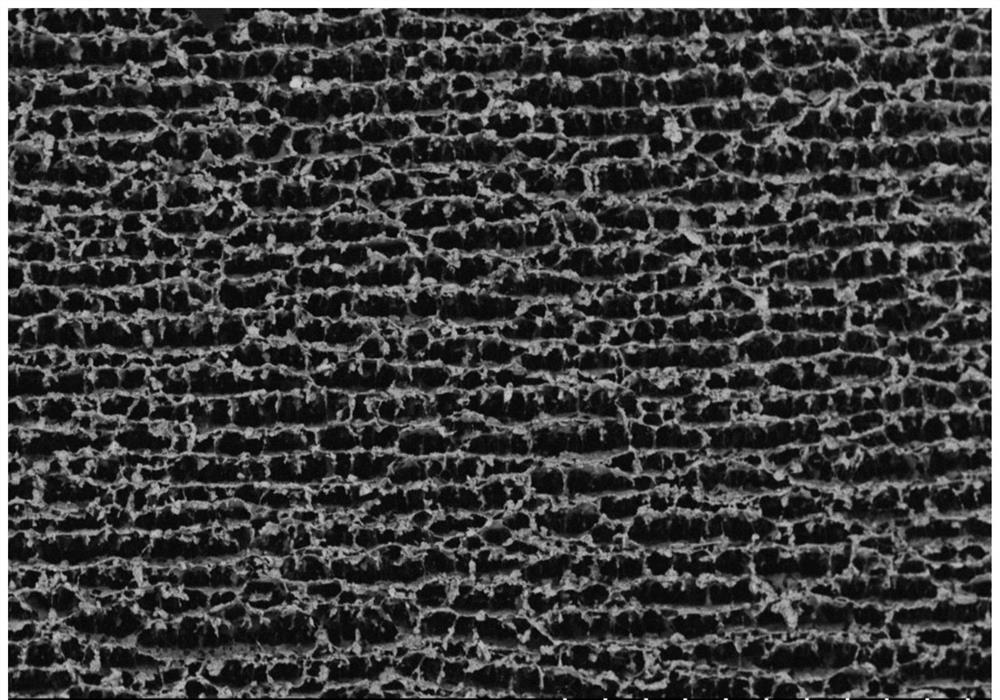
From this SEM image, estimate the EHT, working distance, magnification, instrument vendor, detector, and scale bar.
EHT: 5 kV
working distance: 8.2 mm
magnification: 0.13 K X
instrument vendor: Hitachi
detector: BSE-COMP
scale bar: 400000 nm